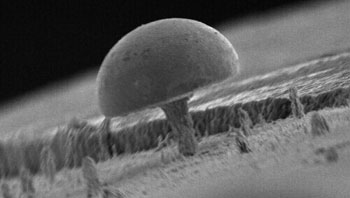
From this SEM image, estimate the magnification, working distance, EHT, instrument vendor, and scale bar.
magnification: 0.5 K X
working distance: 41 mm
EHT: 18 kV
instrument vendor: JEOL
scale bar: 100000 nm